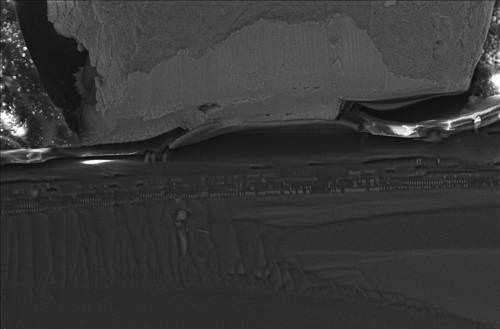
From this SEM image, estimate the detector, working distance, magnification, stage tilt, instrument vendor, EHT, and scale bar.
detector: InLens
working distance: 4 mm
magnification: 2 K X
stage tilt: -15°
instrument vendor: Zeiss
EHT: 15 kV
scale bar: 10000 nm